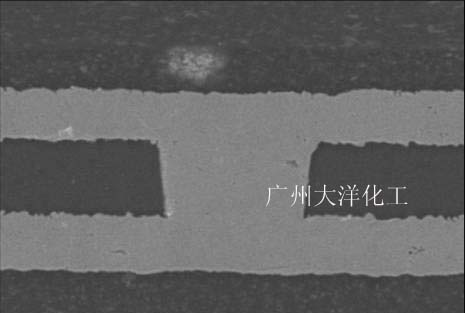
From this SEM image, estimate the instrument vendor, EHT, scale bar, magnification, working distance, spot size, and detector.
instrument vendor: JEOL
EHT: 20 kV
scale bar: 20000 nm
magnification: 0.9 K X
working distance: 23 mm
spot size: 30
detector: SEI